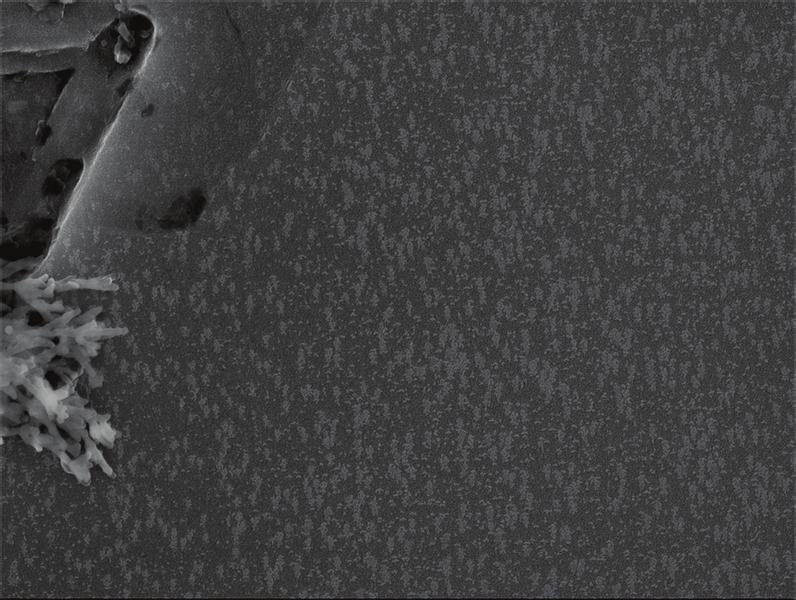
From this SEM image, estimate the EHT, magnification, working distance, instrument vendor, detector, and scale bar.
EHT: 15 kV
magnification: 7 K X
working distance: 14.3 mm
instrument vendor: JEOL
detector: SEI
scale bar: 1000 nm